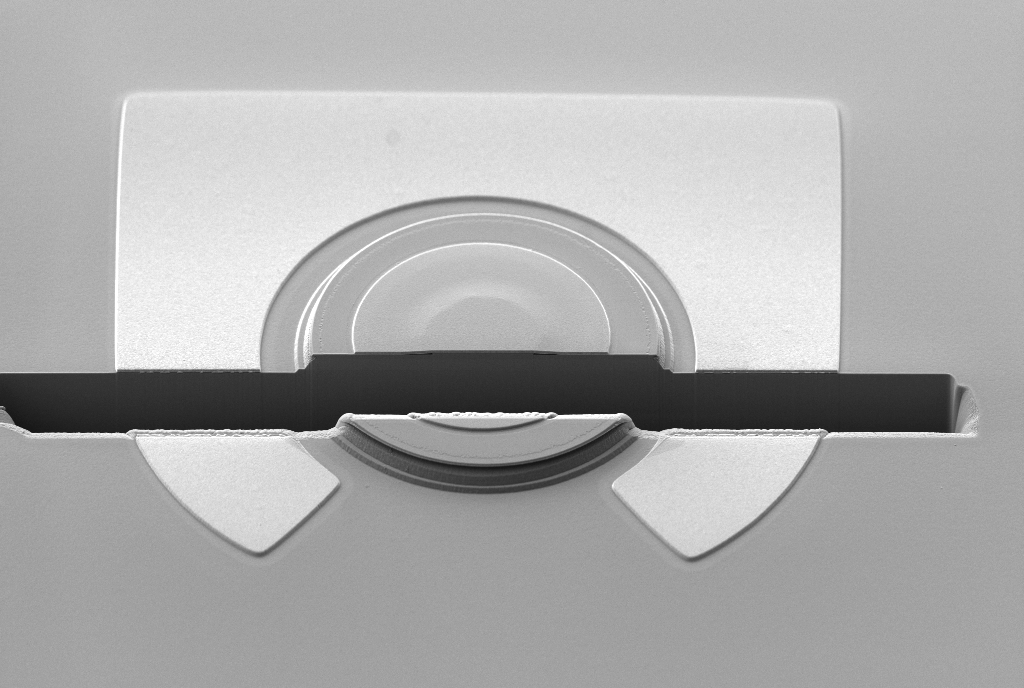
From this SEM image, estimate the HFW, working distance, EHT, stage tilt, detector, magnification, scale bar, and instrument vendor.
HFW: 81.59 µm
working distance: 5.1 mm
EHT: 3 kV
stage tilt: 36°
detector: SE2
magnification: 1.4 K X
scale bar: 10000 nm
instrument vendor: Zeiss